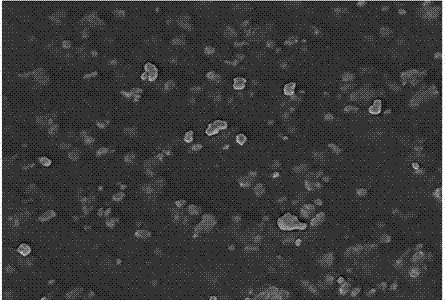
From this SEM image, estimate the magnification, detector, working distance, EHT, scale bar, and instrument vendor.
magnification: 5 K X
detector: SE(U)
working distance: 10.4 mm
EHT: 5 kV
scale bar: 10000 nm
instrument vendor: Hitachi